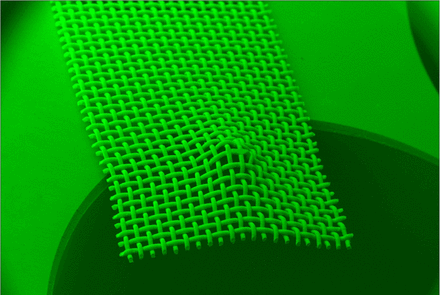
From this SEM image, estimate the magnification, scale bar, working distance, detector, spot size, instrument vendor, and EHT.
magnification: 0.013 K X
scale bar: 1000000 nm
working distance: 37 mm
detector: SEI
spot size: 50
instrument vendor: JEOL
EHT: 30 kV